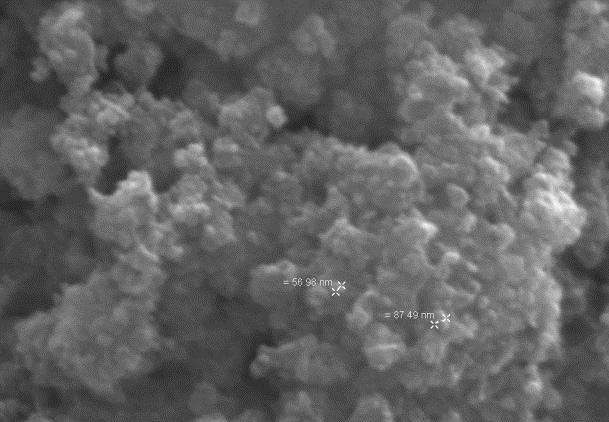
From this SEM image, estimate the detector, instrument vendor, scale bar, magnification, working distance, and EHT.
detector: SE1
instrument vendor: Zeiss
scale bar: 1000 nm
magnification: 80 K X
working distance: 10 mm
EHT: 25 kV